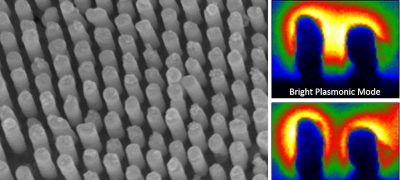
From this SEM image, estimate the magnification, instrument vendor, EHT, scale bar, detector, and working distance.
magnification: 110 K X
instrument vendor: Hitachi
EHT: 15 kV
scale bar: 500 nm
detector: SE(U)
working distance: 6 mm